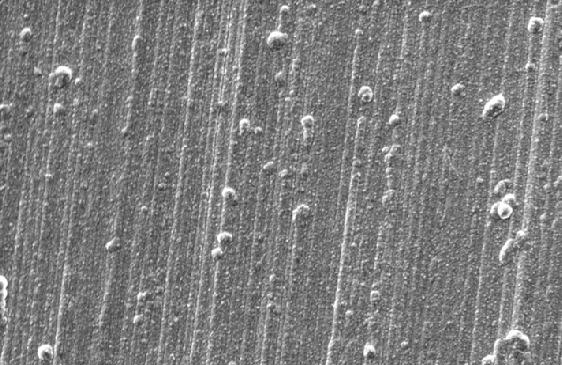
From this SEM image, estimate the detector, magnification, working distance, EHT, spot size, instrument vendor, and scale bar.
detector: SE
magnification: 0.4 K X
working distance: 10.1 mm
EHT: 20 kV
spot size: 5.5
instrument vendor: FEI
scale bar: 50000 nm